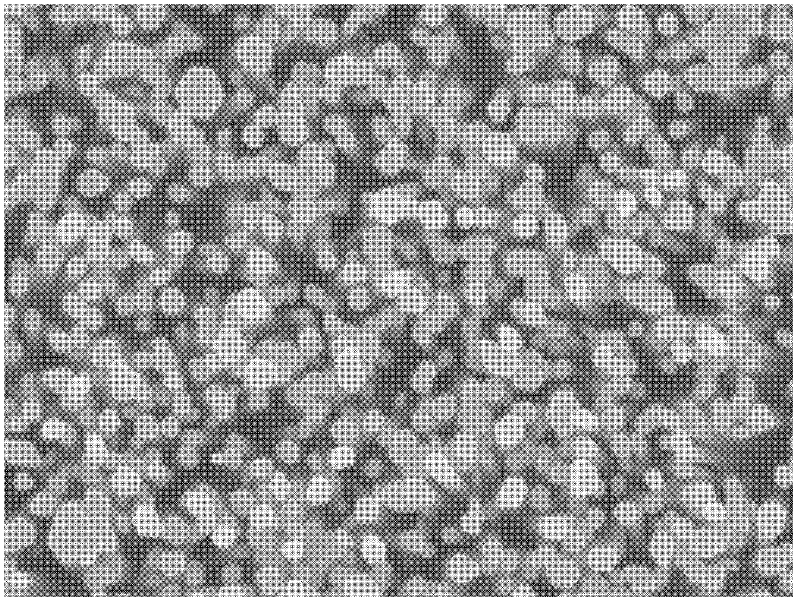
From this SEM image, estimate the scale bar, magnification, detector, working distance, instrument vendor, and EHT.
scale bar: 100 nm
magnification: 30 K X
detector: SEI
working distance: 6.8 mm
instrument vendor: JEOL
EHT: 10 kV